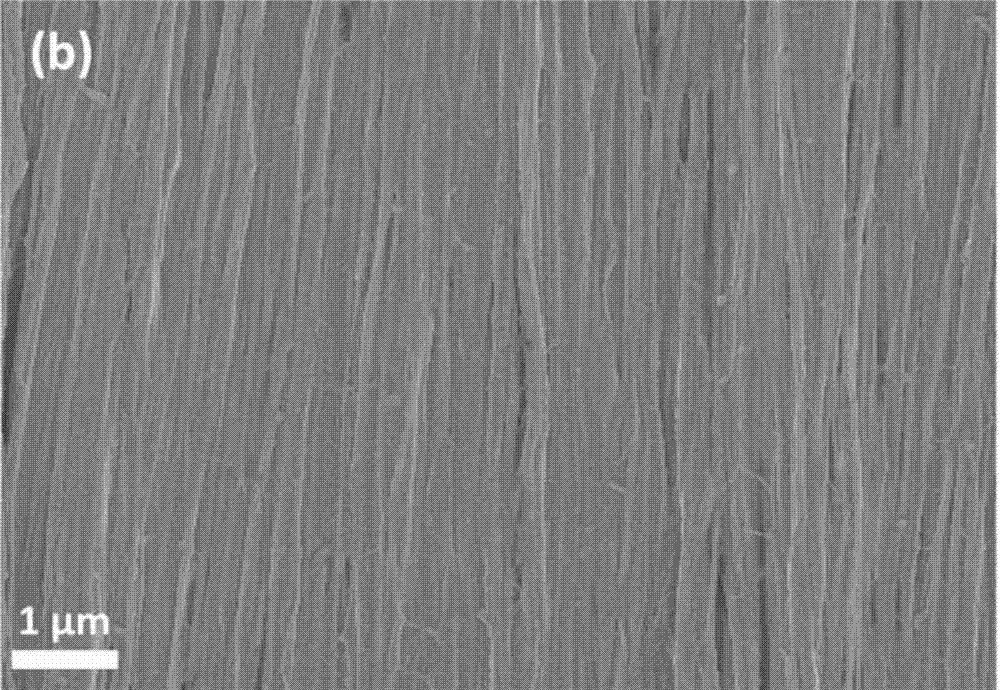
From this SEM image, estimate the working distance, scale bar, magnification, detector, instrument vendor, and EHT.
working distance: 2.2 mm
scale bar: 1000 nm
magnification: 30 K X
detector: InLens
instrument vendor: Zeiss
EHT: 1.1 kV